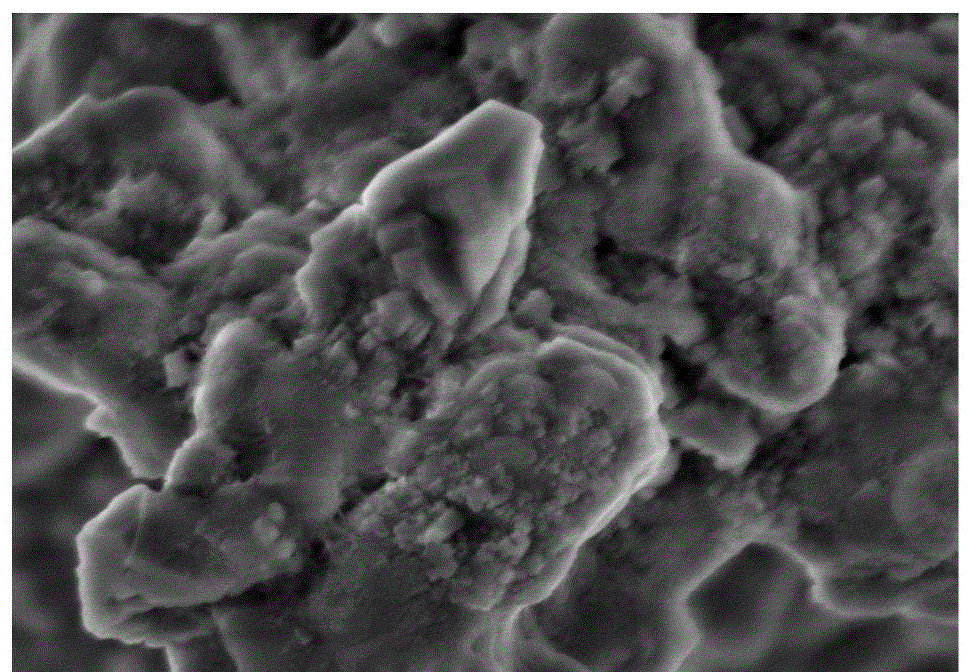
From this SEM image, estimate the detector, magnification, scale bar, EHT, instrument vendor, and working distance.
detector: SE(M)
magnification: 50 K X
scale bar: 1000 nm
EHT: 3 kV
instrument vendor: Hitachi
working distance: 8.7 mm